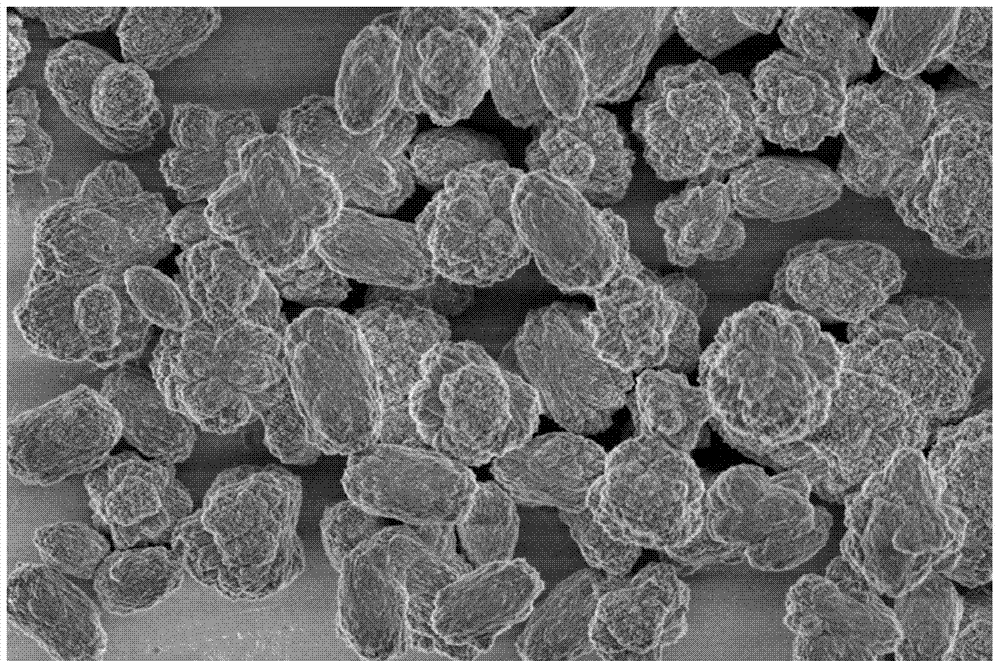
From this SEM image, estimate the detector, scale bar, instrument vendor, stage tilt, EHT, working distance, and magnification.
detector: TLD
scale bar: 4000 nm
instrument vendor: FEI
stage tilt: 0°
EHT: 1 kV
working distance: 4.1 mm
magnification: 30 K X